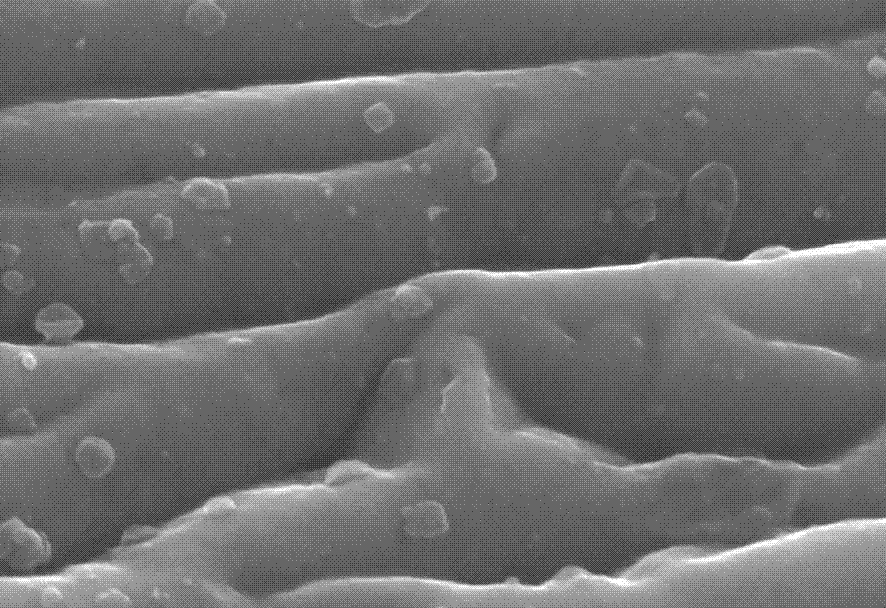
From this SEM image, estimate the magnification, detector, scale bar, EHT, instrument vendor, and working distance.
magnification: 80 K X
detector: SE(U)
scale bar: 500 nm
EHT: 15 kV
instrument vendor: Hitachi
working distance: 9 mm